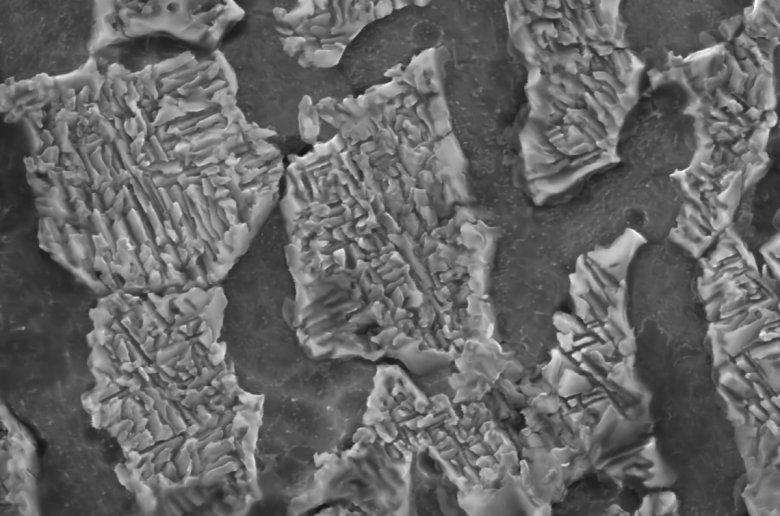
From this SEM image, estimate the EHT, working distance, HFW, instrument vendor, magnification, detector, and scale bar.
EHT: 20 kV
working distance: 8 mm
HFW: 13.55 µm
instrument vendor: FEI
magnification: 30 K X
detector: ETD SE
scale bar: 1000 nm